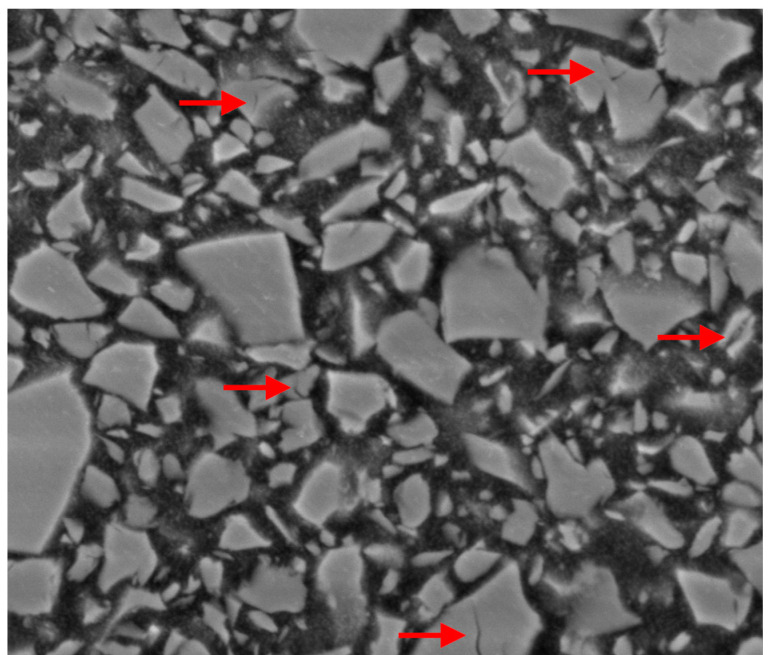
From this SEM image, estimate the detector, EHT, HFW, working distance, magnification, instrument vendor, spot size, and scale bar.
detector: BSE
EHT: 8 kV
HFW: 6.76 µm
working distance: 9.2 mm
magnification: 40 K X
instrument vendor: FEI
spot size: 3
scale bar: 1000 nm